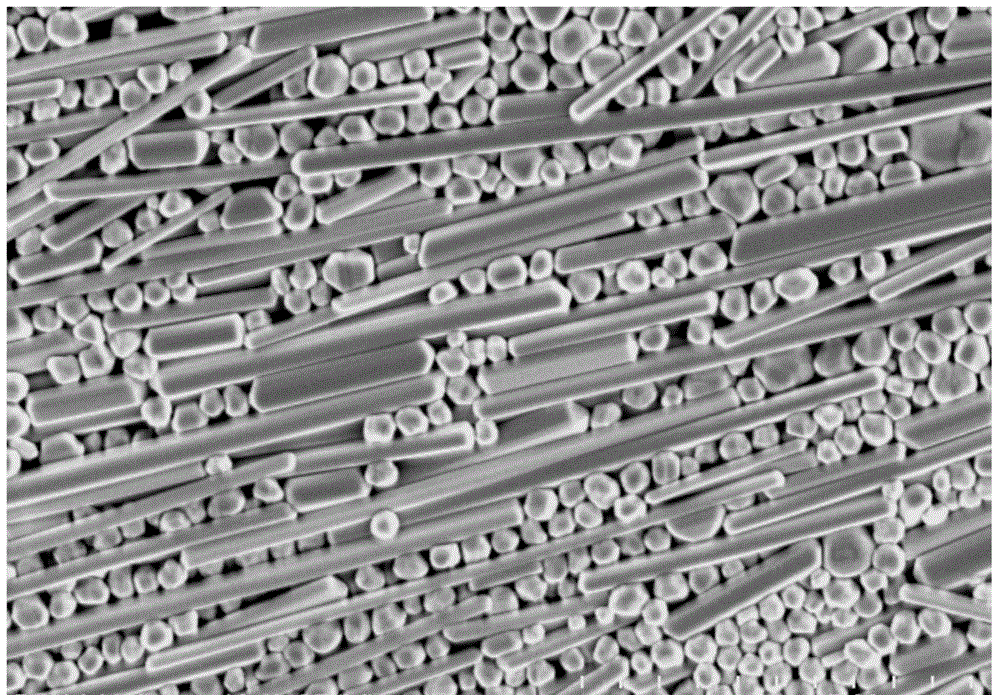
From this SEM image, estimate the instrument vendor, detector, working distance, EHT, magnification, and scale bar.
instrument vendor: Hitachi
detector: SE(M)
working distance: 13.1 mm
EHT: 5 kV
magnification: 10 K X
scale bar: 5000 nm